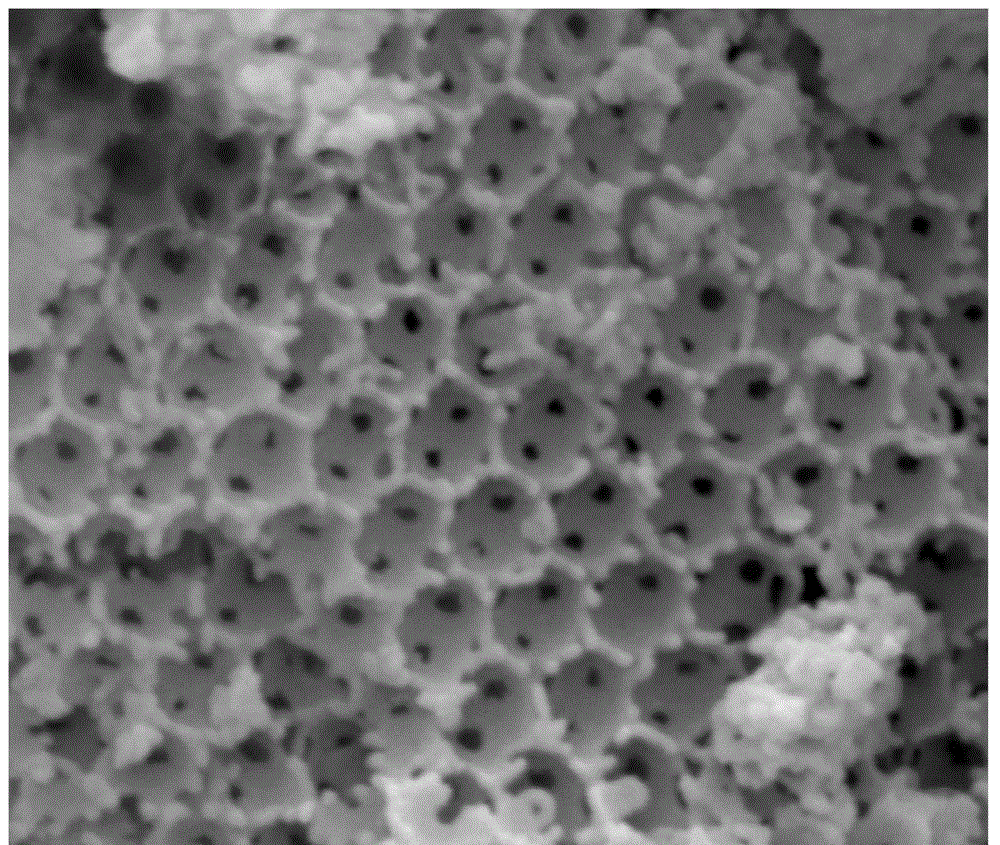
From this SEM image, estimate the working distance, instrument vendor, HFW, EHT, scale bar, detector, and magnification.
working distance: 11.8 mm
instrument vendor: FEI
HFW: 2.56 µm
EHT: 20 kV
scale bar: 1000 nm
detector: ETD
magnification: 100 K X